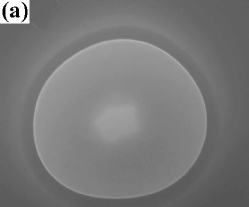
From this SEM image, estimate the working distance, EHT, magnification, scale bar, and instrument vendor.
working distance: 11.2 mm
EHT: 30 kV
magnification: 19.789 K X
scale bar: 2000 nm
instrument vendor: FEI